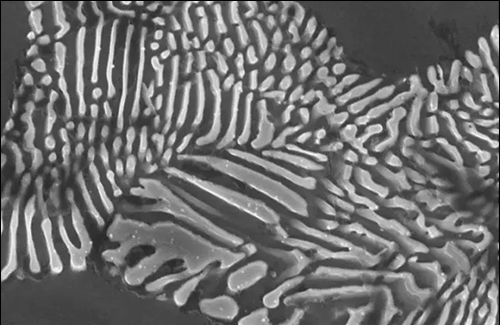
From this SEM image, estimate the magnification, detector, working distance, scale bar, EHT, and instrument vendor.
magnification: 20 K X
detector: SE1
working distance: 9.5 mm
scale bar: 1000 nm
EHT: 20 kV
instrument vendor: Zeiss